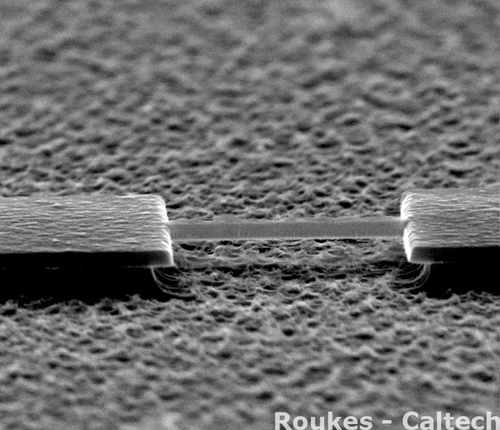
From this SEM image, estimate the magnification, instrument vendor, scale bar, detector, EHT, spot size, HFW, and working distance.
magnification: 40 K X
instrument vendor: FEI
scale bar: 1000 nm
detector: ETD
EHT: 30 kV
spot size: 1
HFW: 3.38 µm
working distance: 8.9 mm